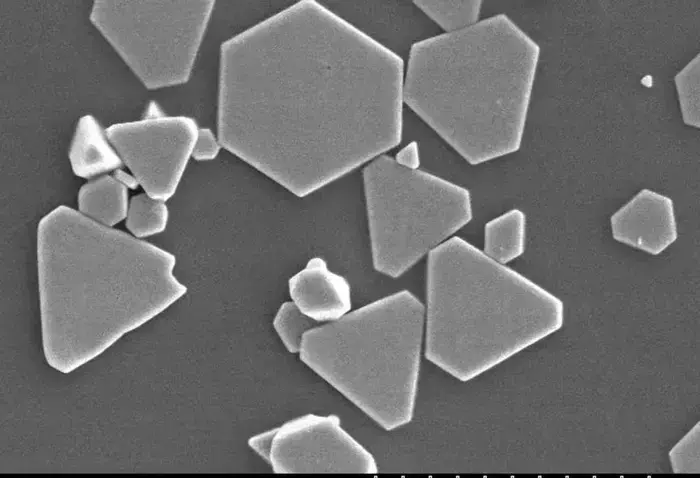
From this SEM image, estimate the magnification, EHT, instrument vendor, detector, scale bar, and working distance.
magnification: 5 K X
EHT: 10 kV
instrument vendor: JEOL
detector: SE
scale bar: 10000 nm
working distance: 12.7 mm